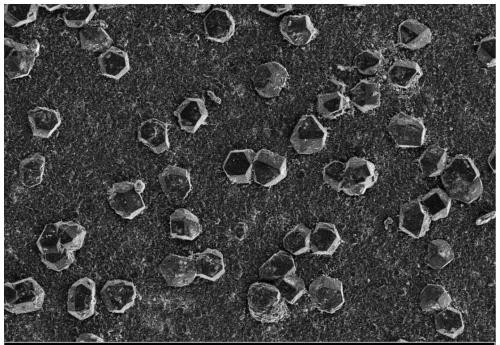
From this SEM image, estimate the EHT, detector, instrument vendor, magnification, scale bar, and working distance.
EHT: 5 kV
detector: SE2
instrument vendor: Zeiss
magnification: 0.5 K X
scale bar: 10000 nm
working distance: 7.8 mm